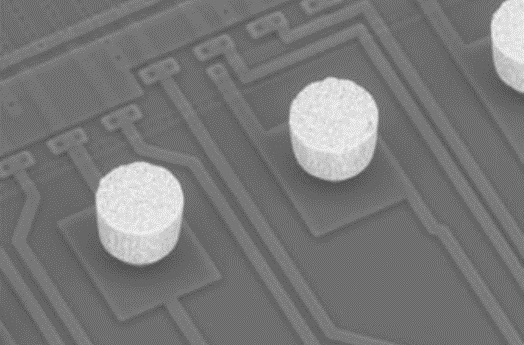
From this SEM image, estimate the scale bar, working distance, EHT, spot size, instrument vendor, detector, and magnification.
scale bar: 20000 nm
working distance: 7.5 mm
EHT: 25 kV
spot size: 6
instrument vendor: FEI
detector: NONE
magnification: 0.75 K X